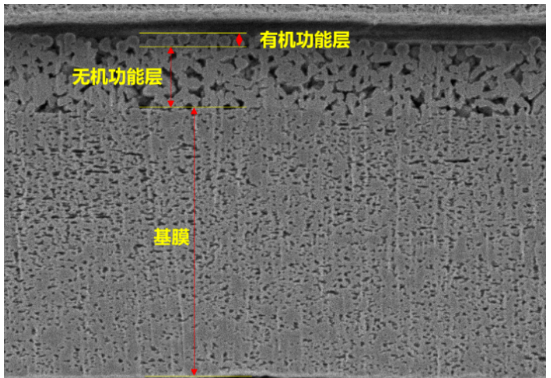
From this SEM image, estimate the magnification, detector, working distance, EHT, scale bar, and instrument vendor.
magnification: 7 K X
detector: SE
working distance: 5.9 mm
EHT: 2 kV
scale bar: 5000 nm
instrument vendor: Hitachi